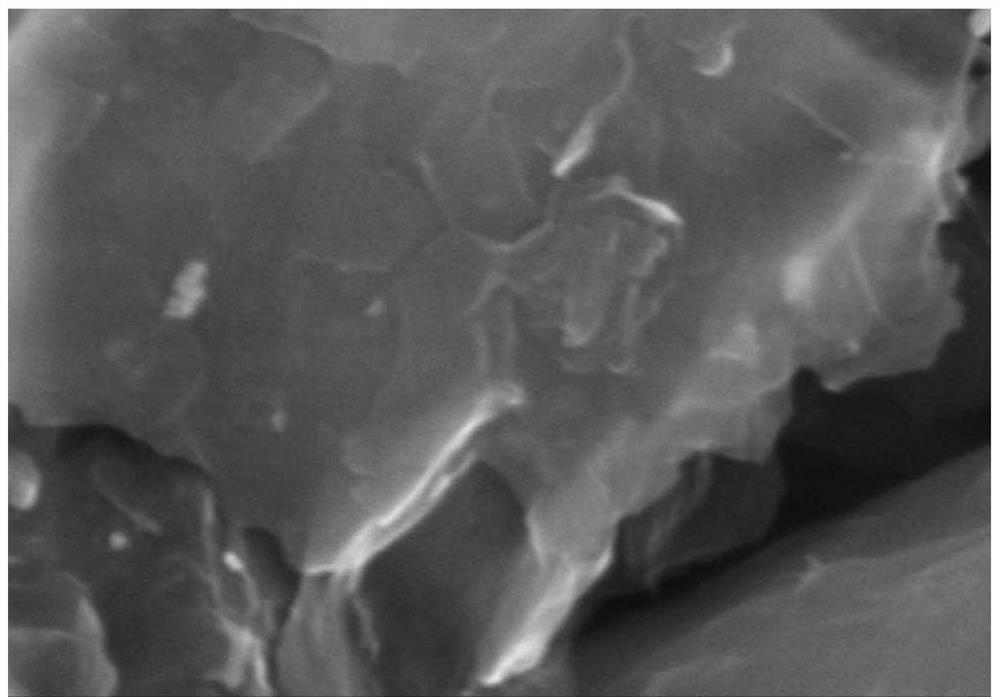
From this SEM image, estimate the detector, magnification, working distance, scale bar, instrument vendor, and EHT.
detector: SE(M)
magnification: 100 K X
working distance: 14.1 mm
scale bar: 500 nm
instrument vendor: Hitachi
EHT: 15 kV